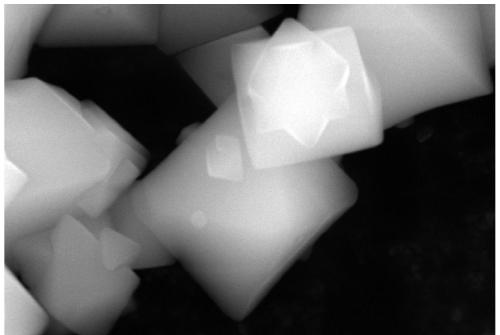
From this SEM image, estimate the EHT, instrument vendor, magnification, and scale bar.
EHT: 20 kV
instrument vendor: JEOL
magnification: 15 K X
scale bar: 1000 nm